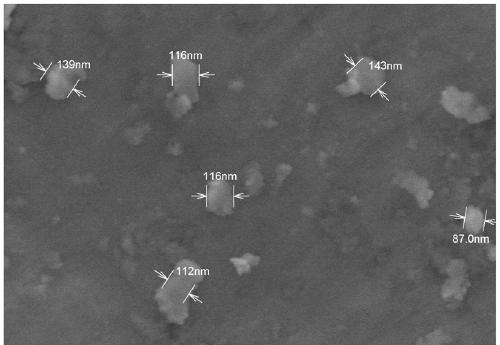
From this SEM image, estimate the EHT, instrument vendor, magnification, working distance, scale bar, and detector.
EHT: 3 kV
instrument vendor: Hitachi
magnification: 60 K X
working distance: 8.4 mm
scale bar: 500 nm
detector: SE(M,LA5)